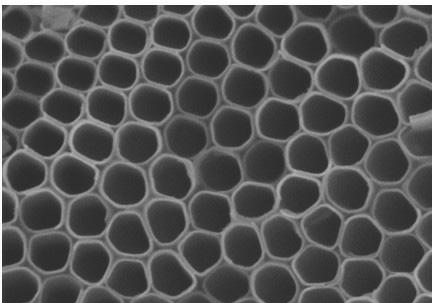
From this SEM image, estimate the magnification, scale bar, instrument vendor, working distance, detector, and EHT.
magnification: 100 K X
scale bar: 500 nm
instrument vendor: Hitachi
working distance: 8 mm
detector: SE(M)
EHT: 10 kV